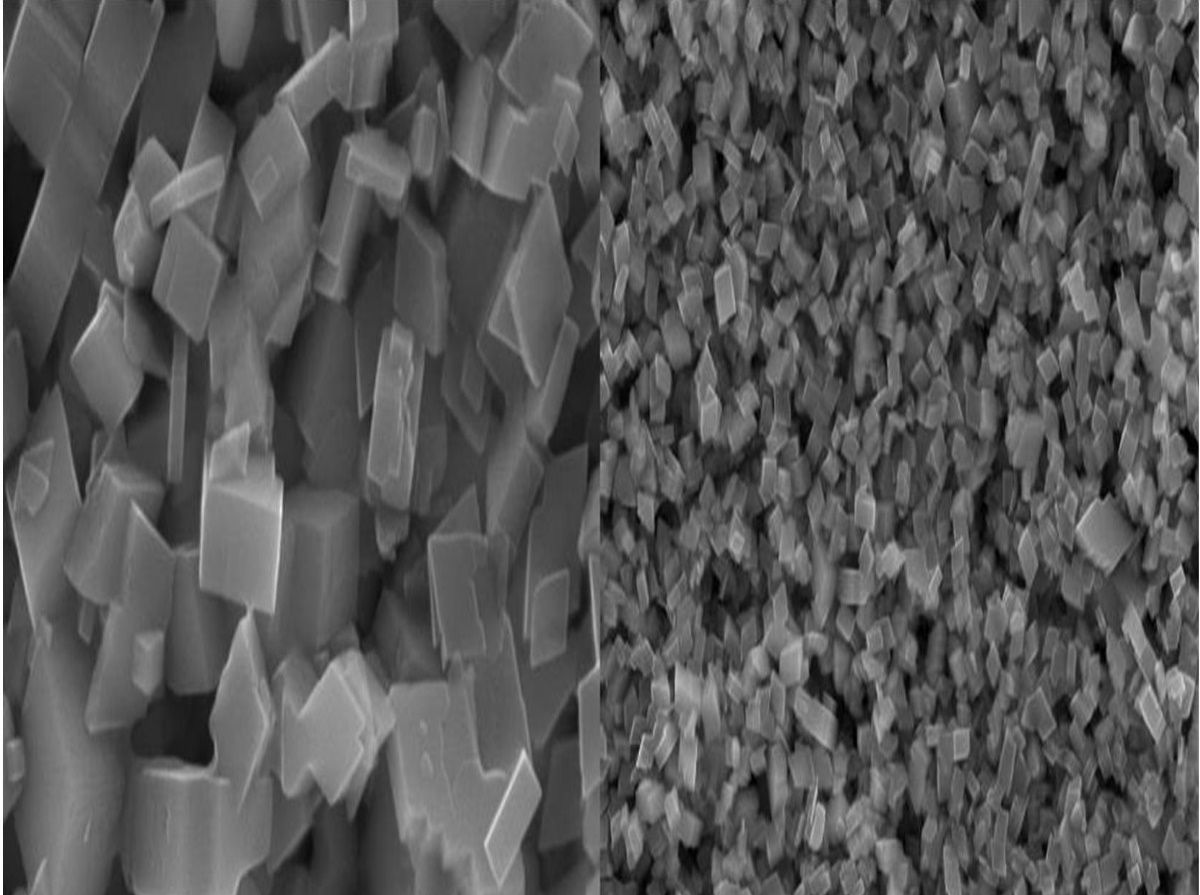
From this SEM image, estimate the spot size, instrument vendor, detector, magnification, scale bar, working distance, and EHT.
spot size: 30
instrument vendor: JEOL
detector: SEI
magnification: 20 K X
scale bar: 1000 nm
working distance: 9 mm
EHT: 20 kV